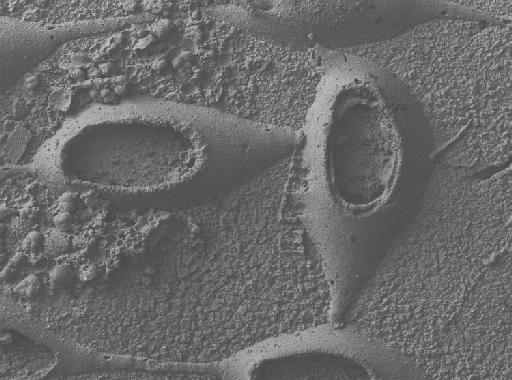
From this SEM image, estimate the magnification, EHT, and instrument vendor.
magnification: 0.066 K X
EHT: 20 kV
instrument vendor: other